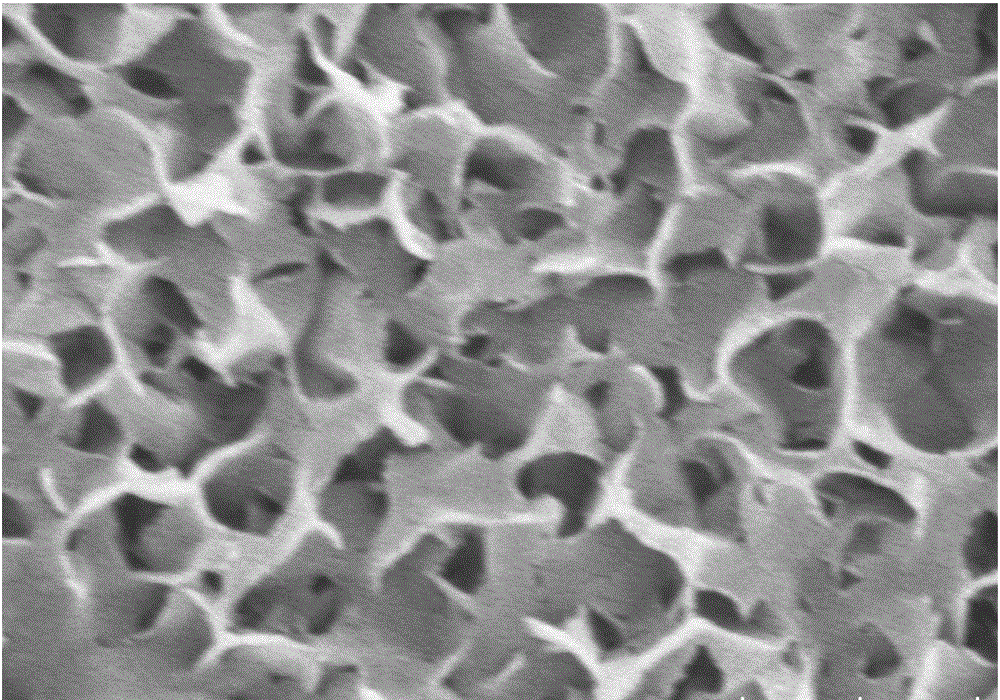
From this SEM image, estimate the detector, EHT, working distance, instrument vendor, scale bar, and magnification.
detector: SE(UL)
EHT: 5 kV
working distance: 9.5 mm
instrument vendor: Hitachi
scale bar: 500 nm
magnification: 60 K X